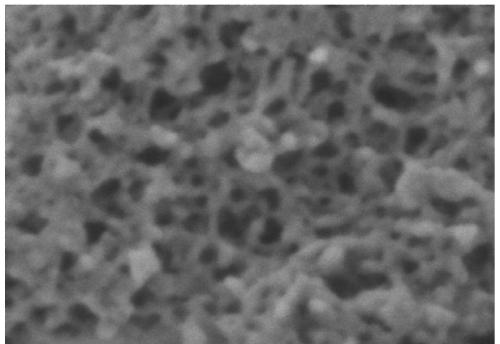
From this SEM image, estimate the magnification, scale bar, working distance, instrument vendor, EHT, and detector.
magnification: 400 K X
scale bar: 20 nm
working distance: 0.9 mm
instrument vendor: Zeiss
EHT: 0.5 kV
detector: InLens